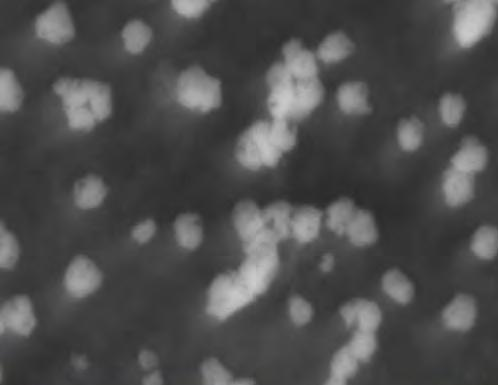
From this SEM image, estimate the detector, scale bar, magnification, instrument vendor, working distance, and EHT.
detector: SE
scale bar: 1000 nm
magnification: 29.471 K X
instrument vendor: Tescan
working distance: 12.1 mm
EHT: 15 kV